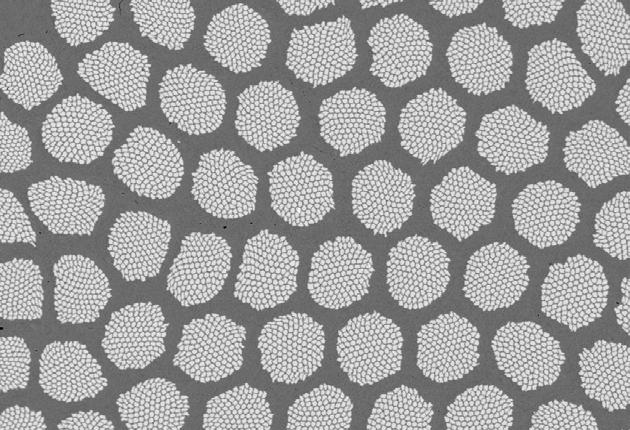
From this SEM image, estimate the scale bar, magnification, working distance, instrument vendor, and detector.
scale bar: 20000 nm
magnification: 0.5 K X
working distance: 14 mm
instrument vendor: Zeiss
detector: QBSD